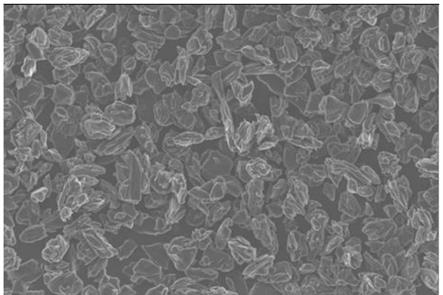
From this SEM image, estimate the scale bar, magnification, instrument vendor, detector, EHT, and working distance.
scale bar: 10000 nm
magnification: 0.5 K X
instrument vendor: Zeiss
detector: InLens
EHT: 10 kV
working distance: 4.3 mm